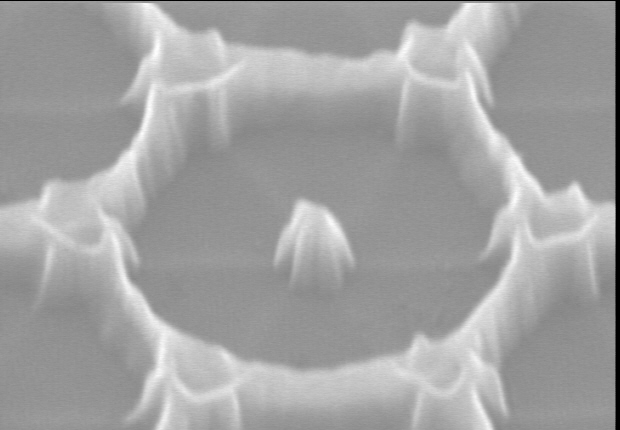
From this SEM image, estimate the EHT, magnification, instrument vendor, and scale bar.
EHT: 10 kV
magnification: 100 K X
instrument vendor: Hitachi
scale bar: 300 nm